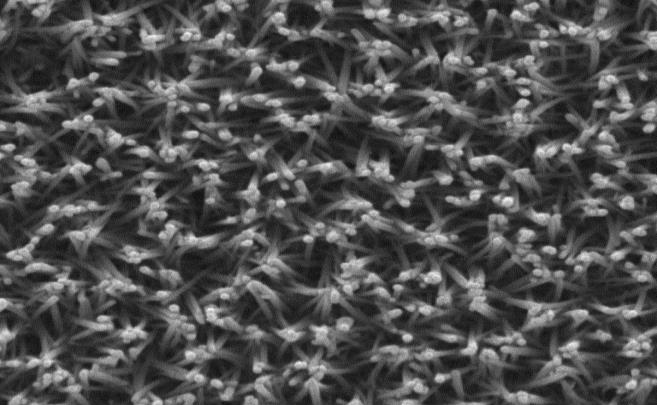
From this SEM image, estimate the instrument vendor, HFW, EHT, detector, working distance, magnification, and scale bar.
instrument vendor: Tescan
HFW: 3.19 µm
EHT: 20 kV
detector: SE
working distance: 8.74 mm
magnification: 65 K X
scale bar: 500 nm